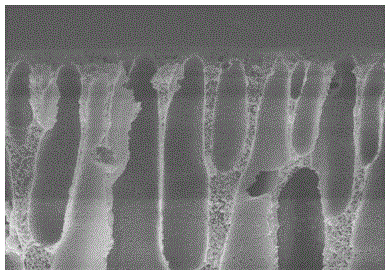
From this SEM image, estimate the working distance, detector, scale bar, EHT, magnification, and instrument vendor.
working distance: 9.2 mm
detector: SE(M)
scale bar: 5000 nm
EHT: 7 kV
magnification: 10 K X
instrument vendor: Hitachi